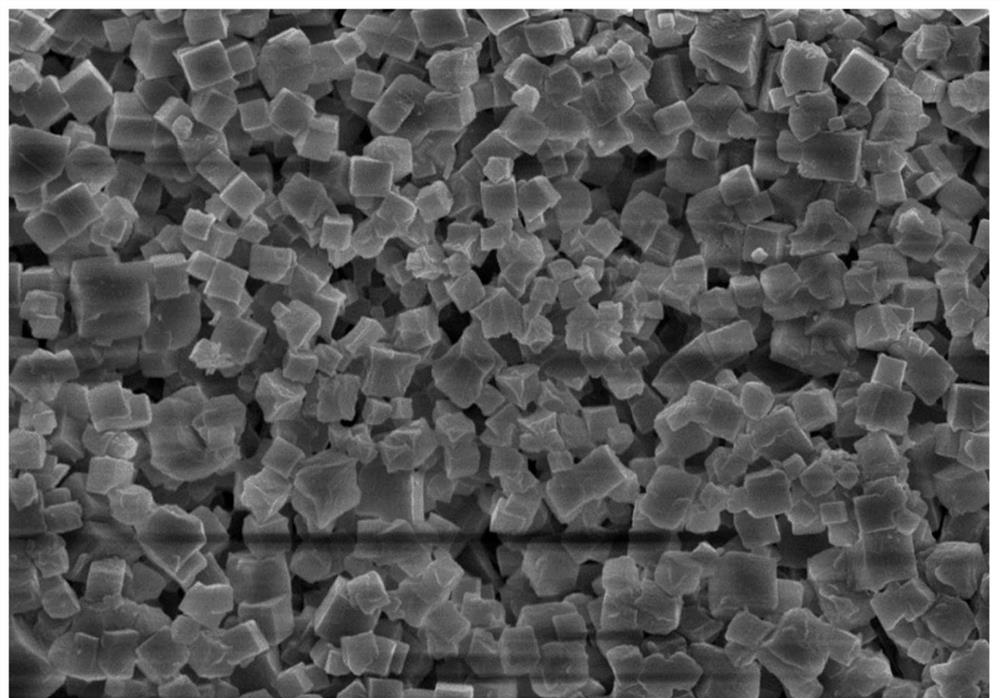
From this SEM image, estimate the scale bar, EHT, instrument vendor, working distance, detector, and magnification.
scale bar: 1000 nm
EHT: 5 kV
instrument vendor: Zeiss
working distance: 4.1 mm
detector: InLens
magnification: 10 K X